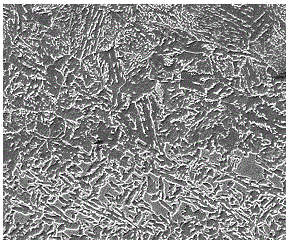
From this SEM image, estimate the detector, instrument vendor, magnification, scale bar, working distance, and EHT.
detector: ETD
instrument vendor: FEI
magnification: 3 K X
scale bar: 10000 nm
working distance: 10.8 mm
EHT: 8 kV